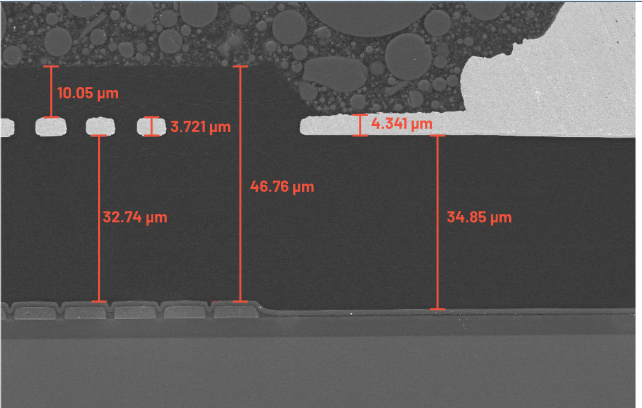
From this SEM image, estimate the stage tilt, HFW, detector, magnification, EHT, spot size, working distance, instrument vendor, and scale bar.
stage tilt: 0°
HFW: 127 µm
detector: ETD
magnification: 1 K X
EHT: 10 kV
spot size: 3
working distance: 10 mm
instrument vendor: FEI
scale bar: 50000 nm